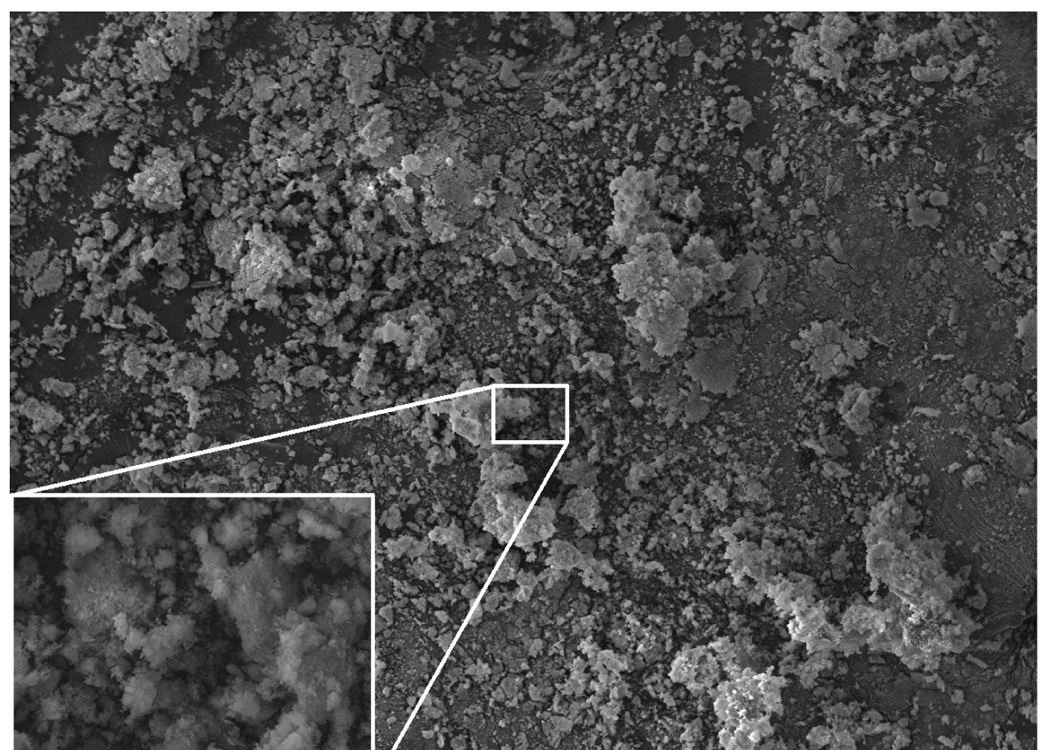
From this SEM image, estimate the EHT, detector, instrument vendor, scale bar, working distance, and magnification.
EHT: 15 kV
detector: SEI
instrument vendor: JEOL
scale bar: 100000 nm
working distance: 9 mm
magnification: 0.1 K X